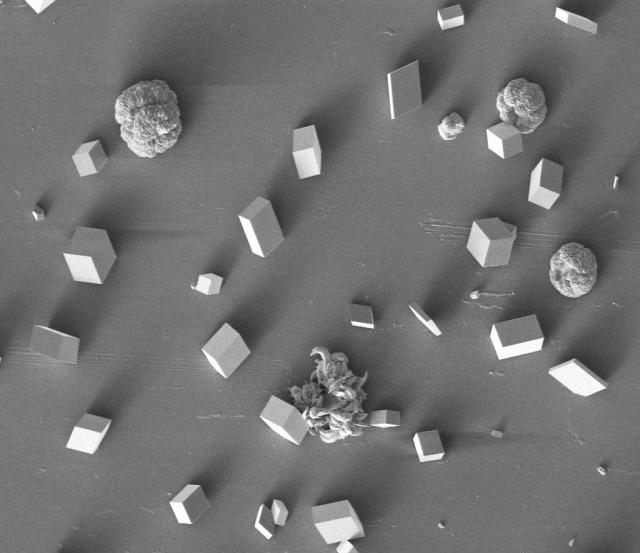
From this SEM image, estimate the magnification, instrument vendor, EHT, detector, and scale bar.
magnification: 0.4 K X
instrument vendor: Tescan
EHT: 5 kV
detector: SE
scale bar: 200000 nm